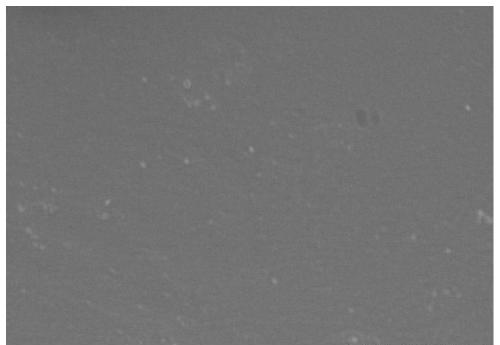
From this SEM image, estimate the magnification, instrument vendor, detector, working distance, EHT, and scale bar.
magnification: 0.7 K X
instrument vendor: Hitachi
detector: SE(U)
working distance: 10.7 mm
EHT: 5 kV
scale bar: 50000 nm